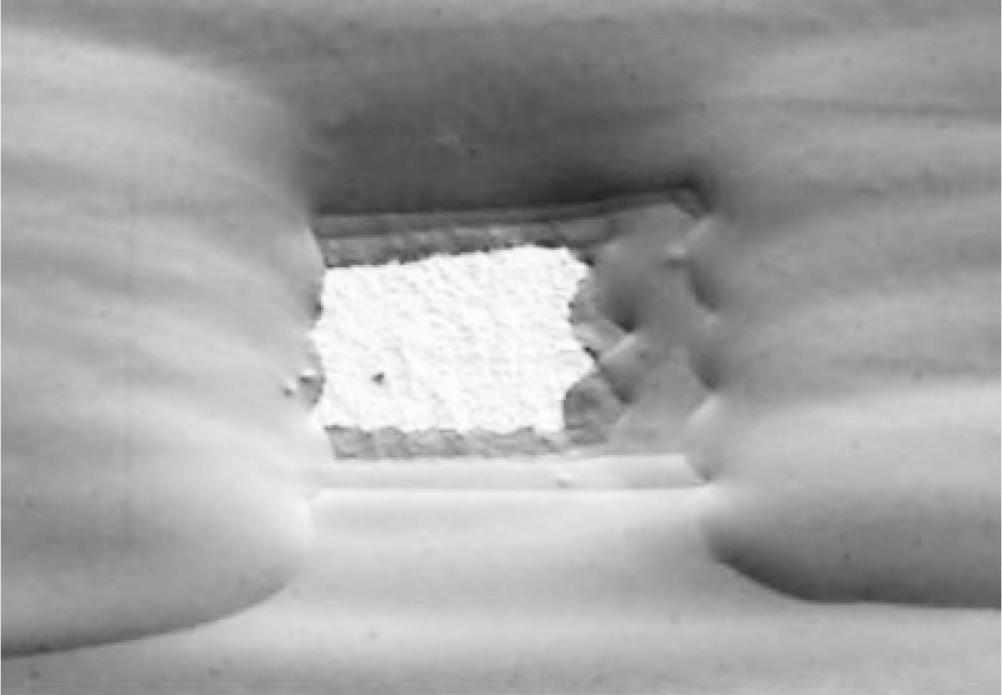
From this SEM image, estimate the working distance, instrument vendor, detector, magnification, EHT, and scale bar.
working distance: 12.6 mm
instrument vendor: Hitachi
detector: SE(L)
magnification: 0.5 K X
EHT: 0.7 kV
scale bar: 100000 nm